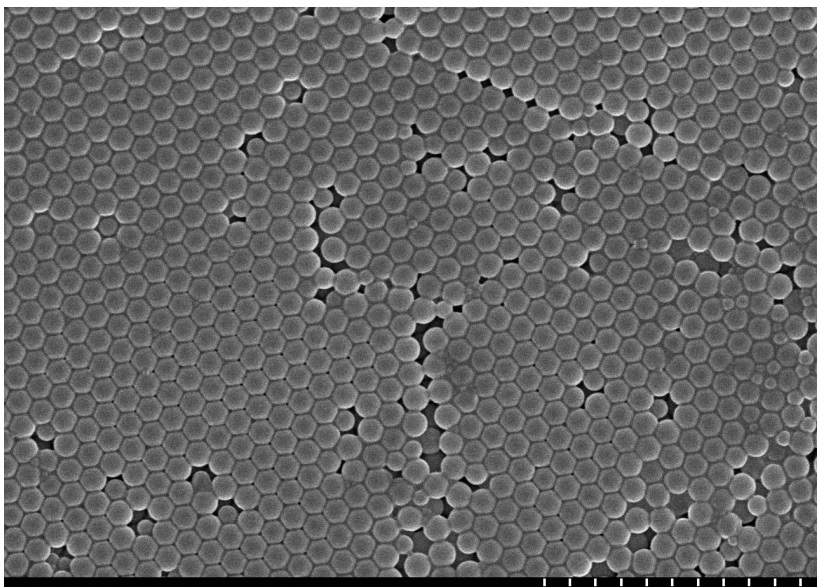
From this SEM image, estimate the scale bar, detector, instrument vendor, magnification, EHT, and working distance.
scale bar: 2000 nm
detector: SE(M)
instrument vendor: Hitachi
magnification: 20 K X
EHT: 10 kV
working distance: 8.6 mm